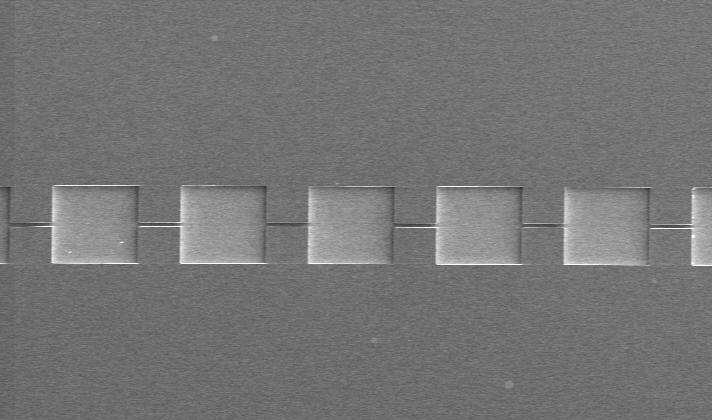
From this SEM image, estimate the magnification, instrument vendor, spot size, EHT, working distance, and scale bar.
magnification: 0.12 K X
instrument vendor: FEI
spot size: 3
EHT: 5 kV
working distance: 10 mm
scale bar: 200000 nm